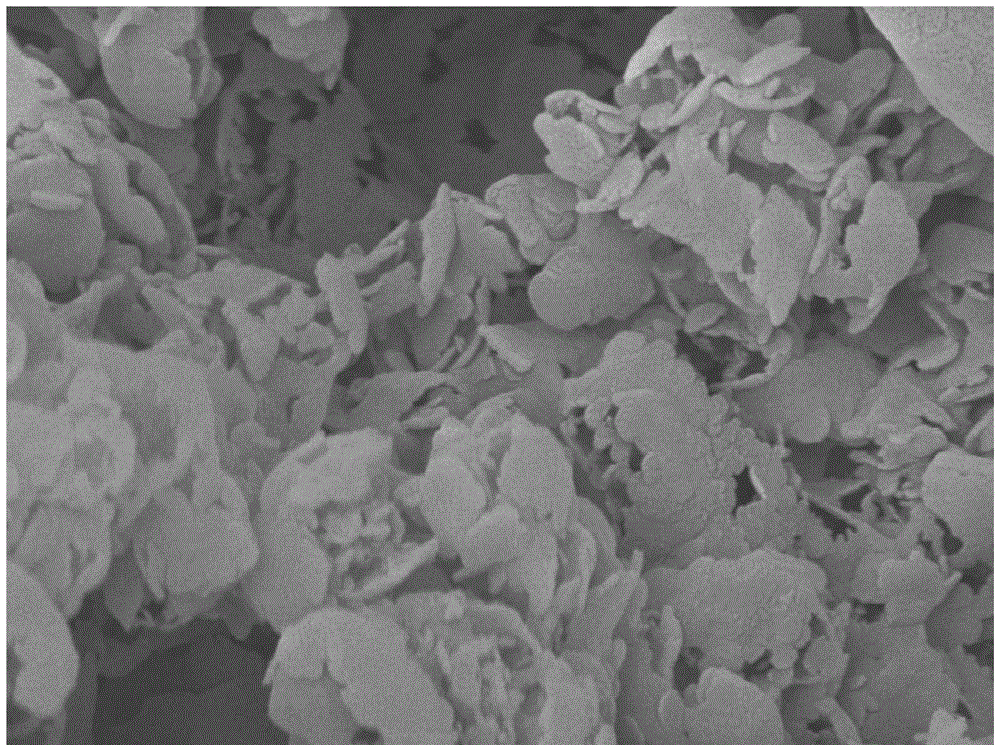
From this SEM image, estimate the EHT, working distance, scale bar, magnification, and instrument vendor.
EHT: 5 kV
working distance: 7.5 mm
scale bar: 100 nm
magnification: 30 K X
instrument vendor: JEOL